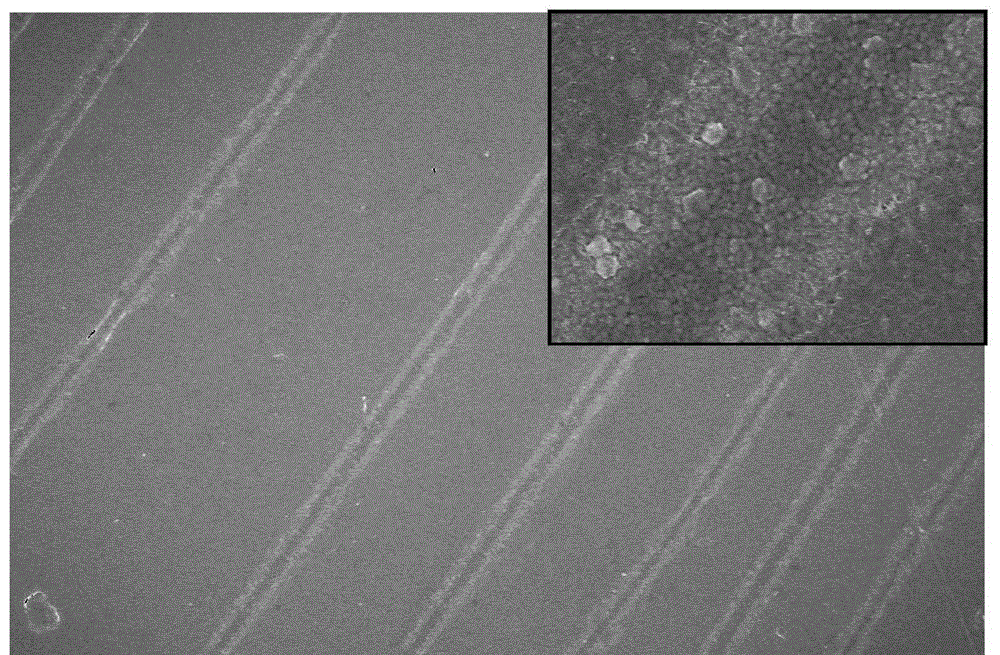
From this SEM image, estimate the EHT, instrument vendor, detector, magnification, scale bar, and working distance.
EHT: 10 kV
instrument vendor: JEOL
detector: SEI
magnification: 1 K X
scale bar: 10000 nm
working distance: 9.2 mm